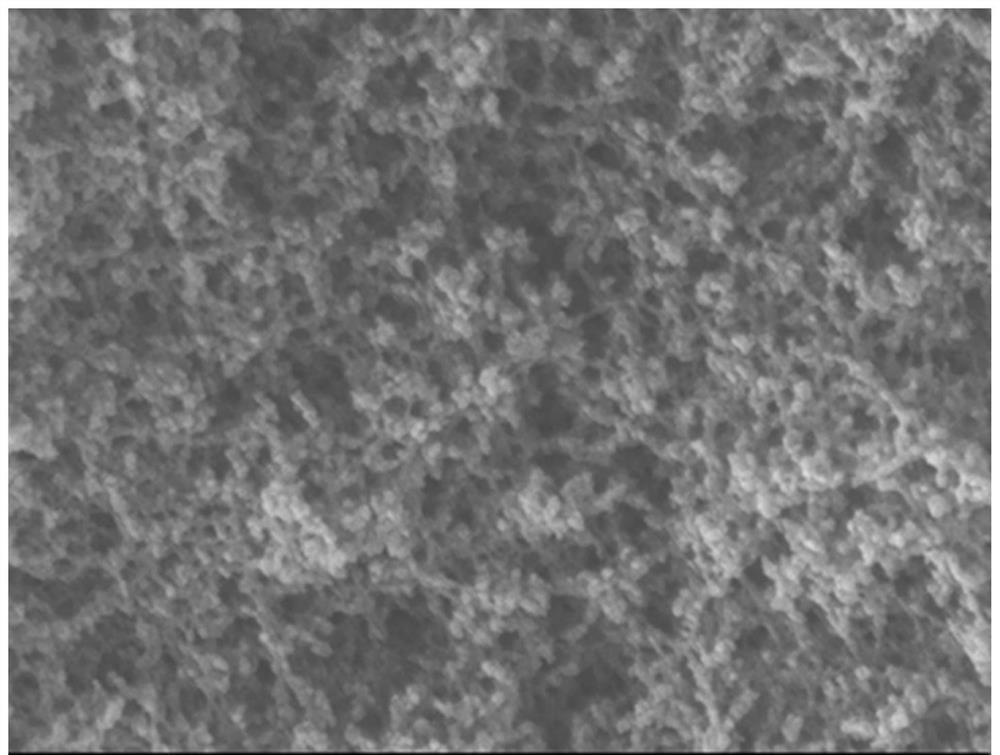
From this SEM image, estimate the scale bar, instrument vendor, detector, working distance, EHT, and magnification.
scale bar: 100 nm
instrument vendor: JEOL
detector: LEI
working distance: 8.5 mm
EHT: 5 kV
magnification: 50 K X